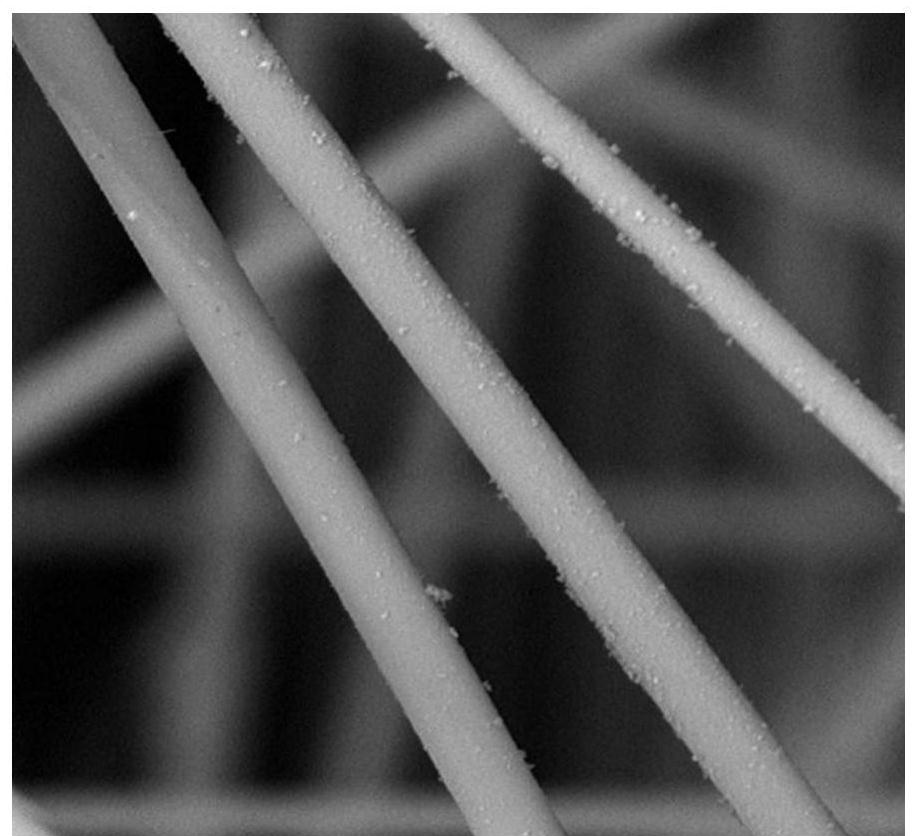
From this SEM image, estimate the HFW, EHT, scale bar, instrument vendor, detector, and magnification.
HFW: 43.7 µm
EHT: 10 kV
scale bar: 10000 nm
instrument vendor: Phenom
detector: BSD Full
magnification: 6.2 K X